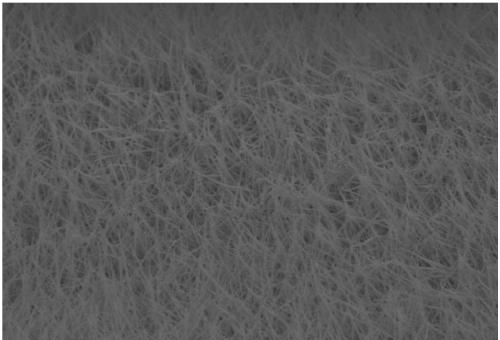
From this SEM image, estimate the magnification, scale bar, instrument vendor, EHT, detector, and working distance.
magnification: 3 K X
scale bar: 10000 nm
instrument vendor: Hitachi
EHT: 5 kV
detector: SE(M)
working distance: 17.5 mm